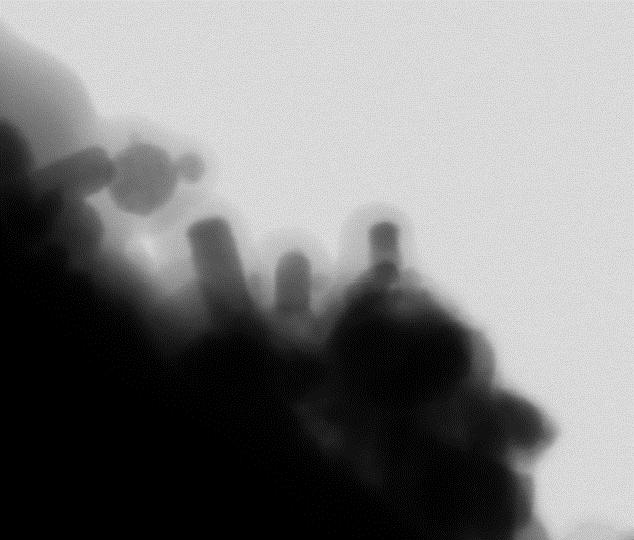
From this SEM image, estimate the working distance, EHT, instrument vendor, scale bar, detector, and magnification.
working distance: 9.8 mm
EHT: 30 kV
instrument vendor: FEI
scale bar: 100 nm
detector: STEM II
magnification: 350 K X